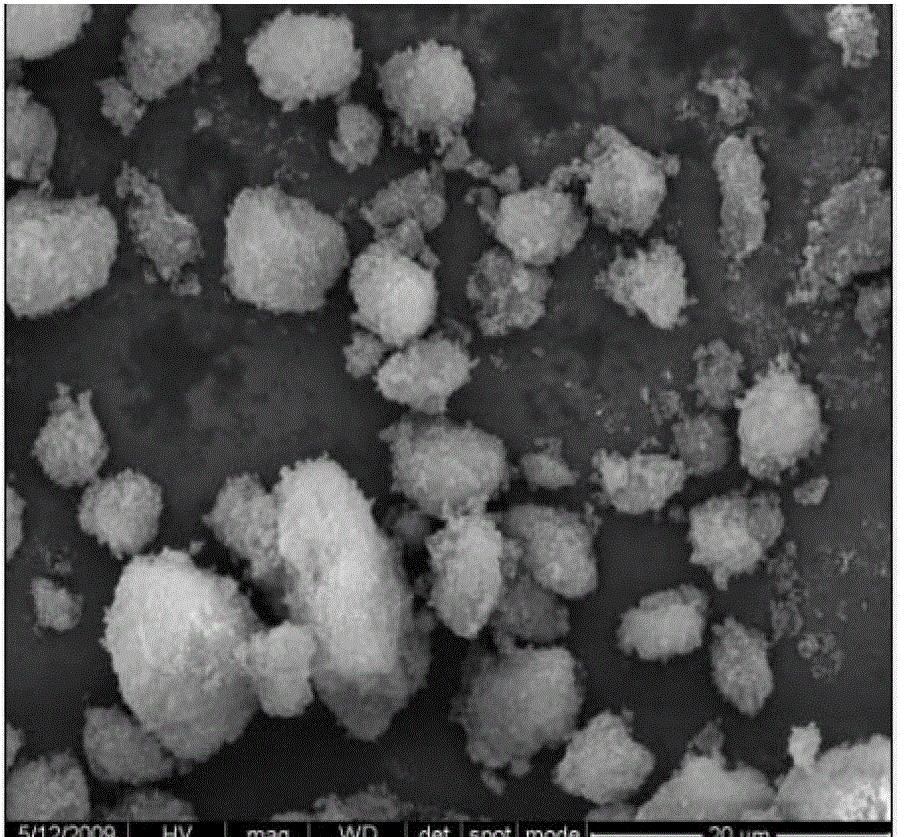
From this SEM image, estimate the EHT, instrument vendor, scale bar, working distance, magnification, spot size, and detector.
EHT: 20 kV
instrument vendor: FEI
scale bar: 20000 nm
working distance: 23.5 mm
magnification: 5 K X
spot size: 4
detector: ETD